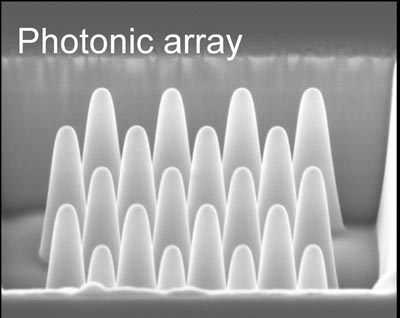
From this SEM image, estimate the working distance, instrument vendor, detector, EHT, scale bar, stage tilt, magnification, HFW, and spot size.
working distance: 4.76 mm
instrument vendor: FEI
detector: TLD-S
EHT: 5 kV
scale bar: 500 nm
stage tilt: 52°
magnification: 120 K X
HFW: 2.53 µm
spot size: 3